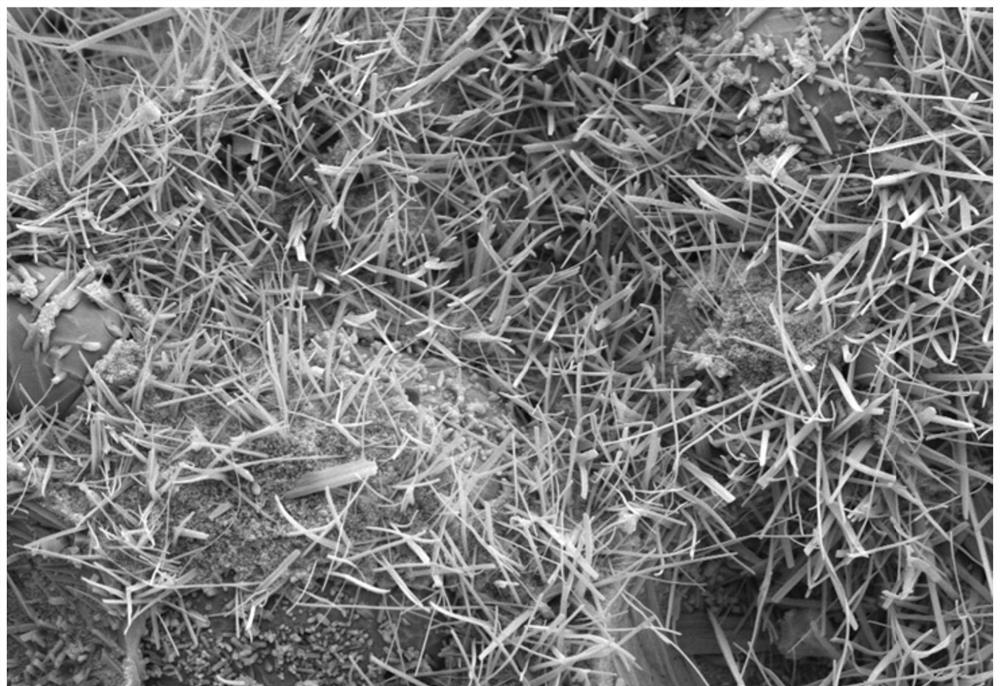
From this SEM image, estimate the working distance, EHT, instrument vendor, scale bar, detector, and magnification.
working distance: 7.7 mm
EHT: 5 kV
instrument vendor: Zeiss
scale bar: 2000 nm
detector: SE2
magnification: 5 K X